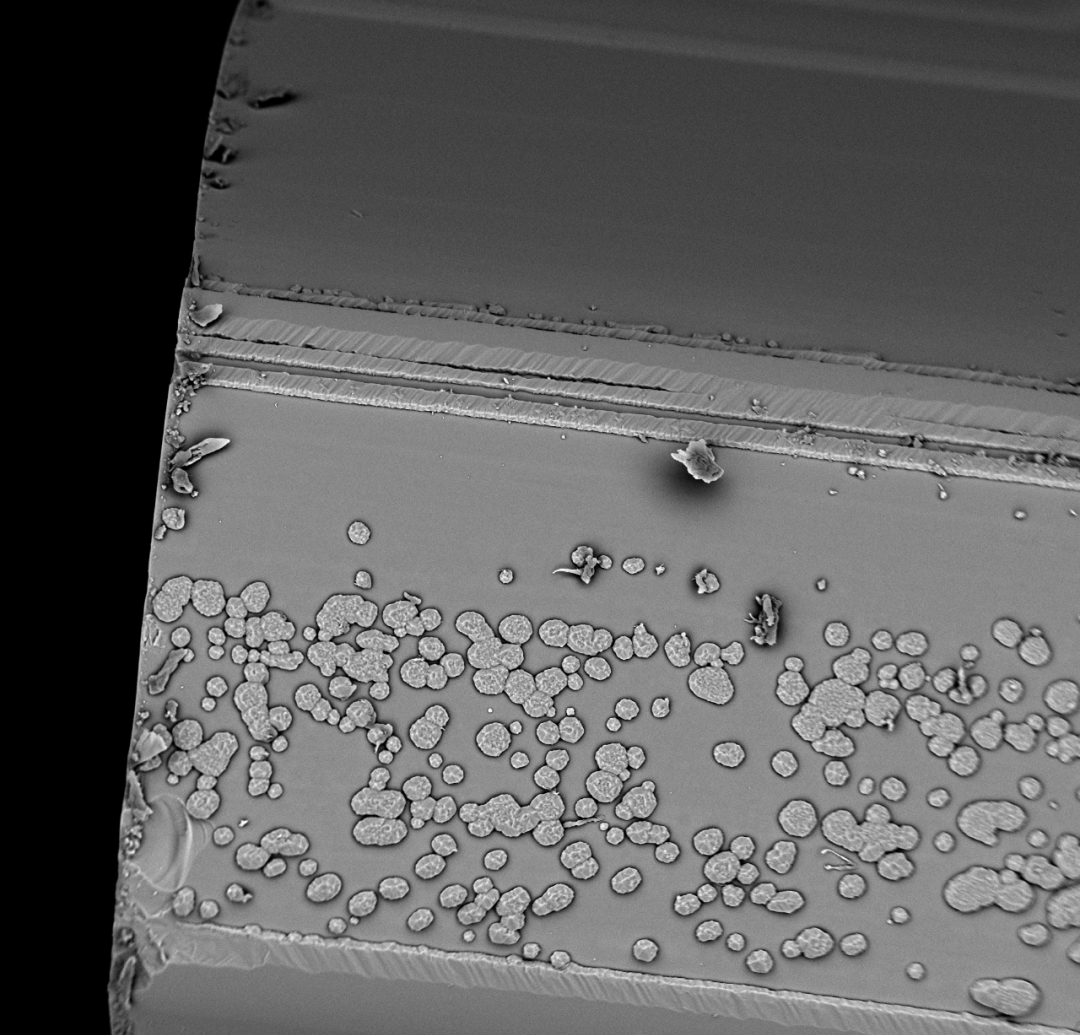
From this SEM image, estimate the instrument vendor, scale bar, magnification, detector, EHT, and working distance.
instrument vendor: other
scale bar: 100000 nm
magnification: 0.4 K X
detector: BSE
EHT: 15 kV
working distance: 6.1 mm